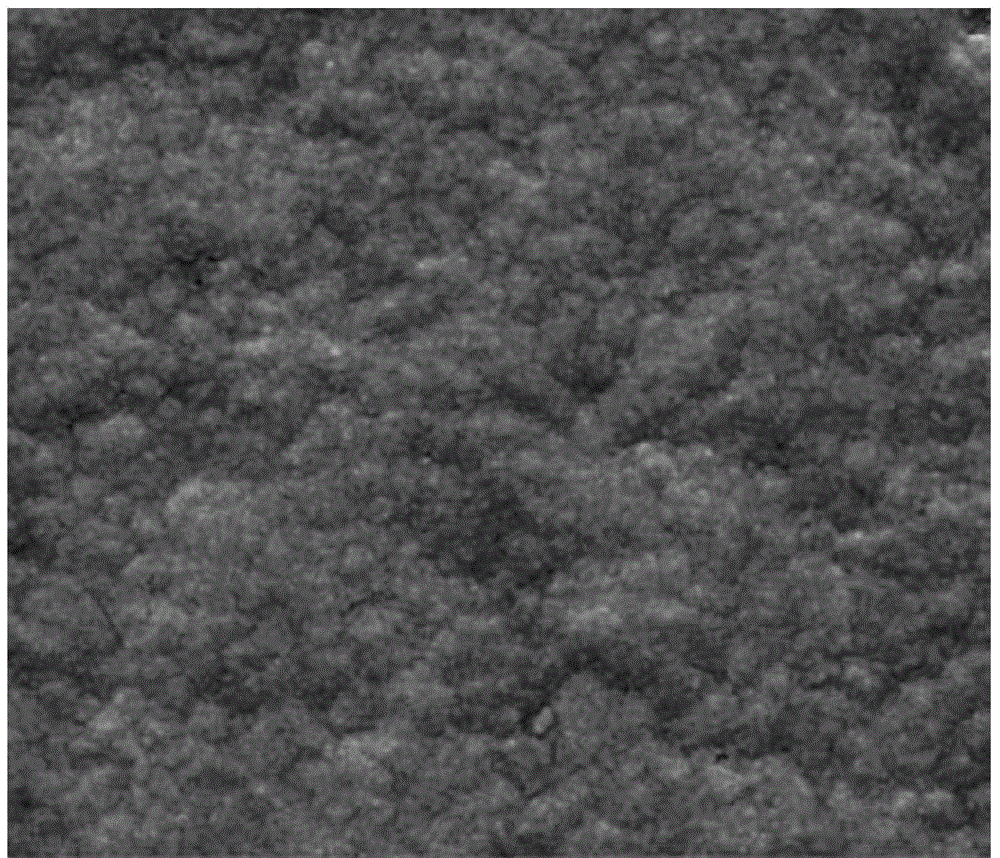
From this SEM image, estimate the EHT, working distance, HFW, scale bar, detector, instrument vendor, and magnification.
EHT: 30 kV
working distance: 12.5 mm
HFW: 2.56 µm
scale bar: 1000 nm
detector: ETD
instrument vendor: FEI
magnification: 100 K X